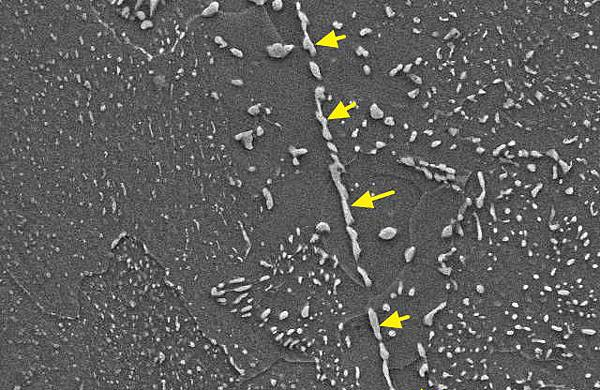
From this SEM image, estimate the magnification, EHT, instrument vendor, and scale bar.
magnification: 2 K X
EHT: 20 kV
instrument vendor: JEOL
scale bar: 10000 nm